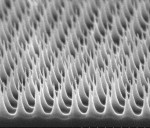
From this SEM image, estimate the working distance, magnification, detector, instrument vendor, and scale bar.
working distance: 5.5 mm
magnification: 50 K X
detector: SE(U)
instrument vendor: Hitachi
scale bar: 1000 nm